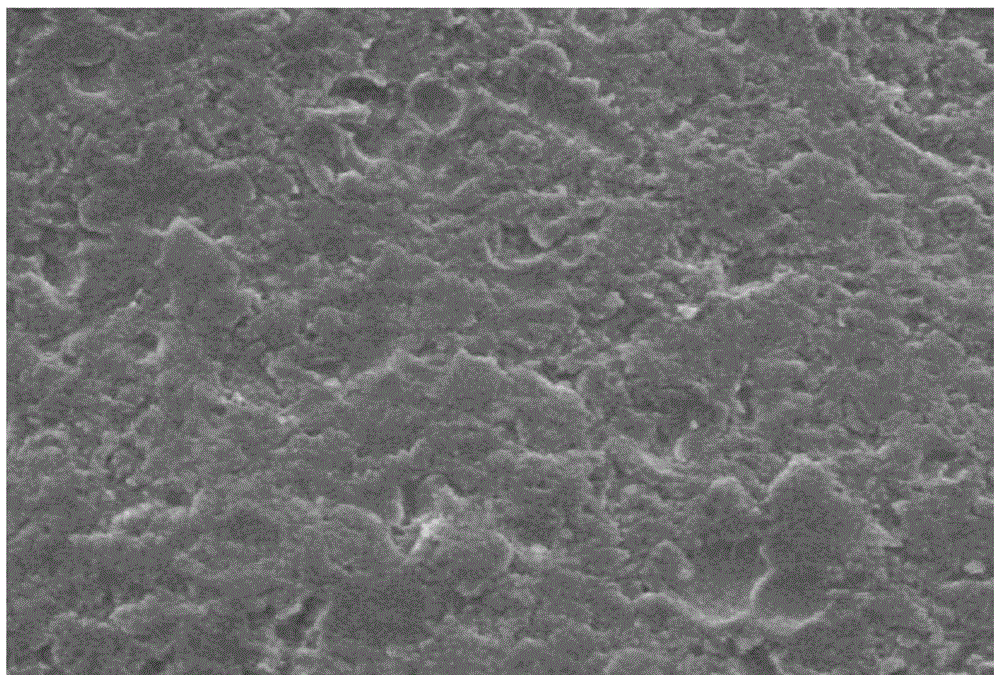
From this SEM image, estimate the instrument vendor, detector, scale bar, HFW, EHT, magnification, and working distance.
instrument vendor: FEI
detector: ETD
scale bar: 20000 nm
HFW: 170 µm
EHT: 20 kV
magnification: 0.8 K X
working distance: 13.9 mm